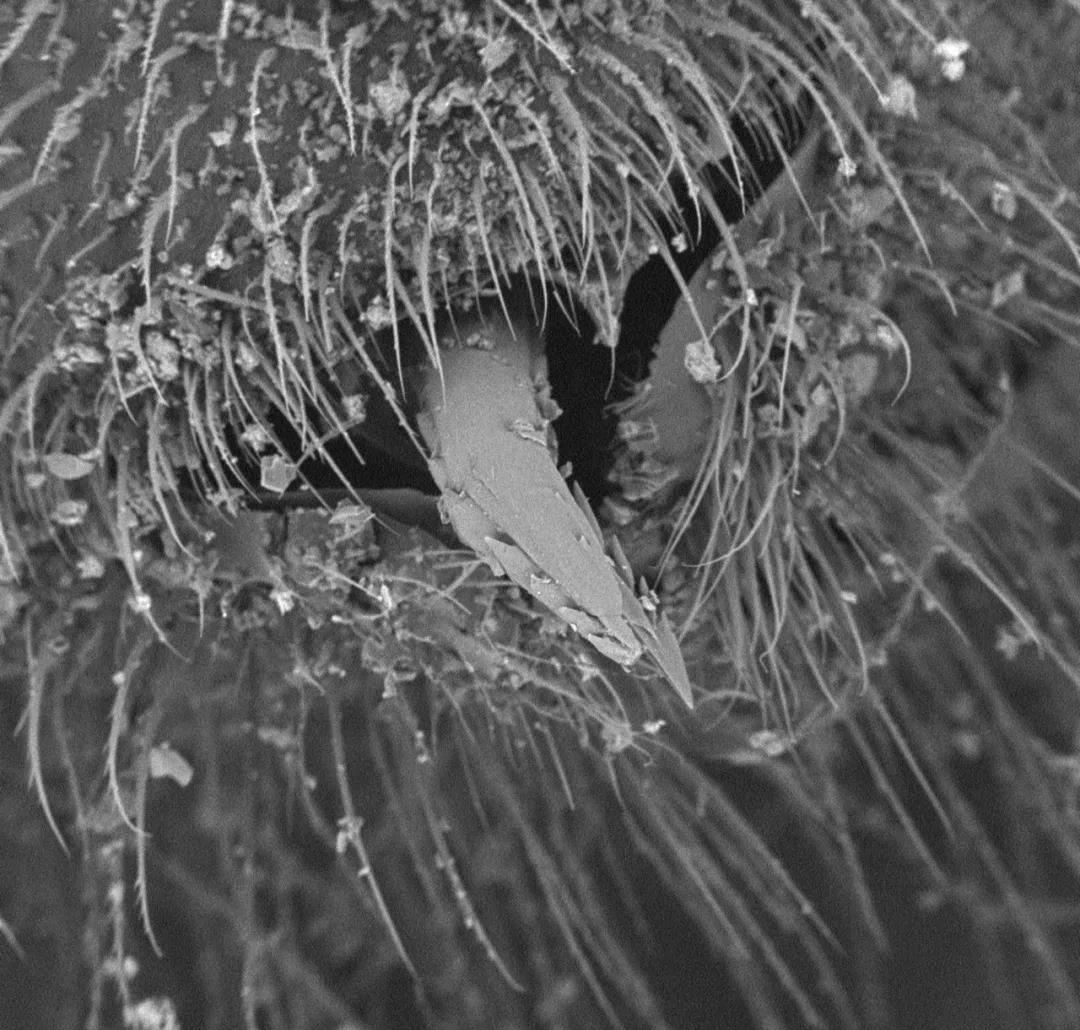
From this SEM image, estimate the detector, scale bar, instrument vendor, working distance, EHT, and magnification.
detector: BSE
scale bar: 100000 nm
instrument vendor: other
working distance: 6.5 mm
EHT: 15 kV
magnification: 0.5 K X